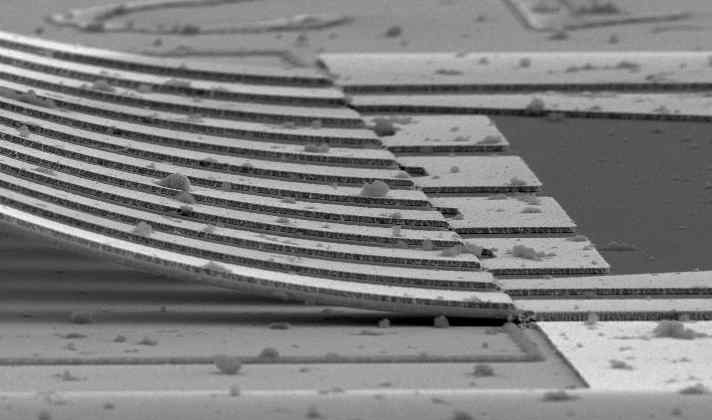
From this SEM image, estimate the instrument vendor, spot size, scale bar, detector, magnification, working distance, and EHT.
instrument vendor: FEI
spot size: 3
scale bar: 10000 nm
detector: SE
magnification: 4.8 K X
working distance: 6.9 mm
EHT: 10 kV